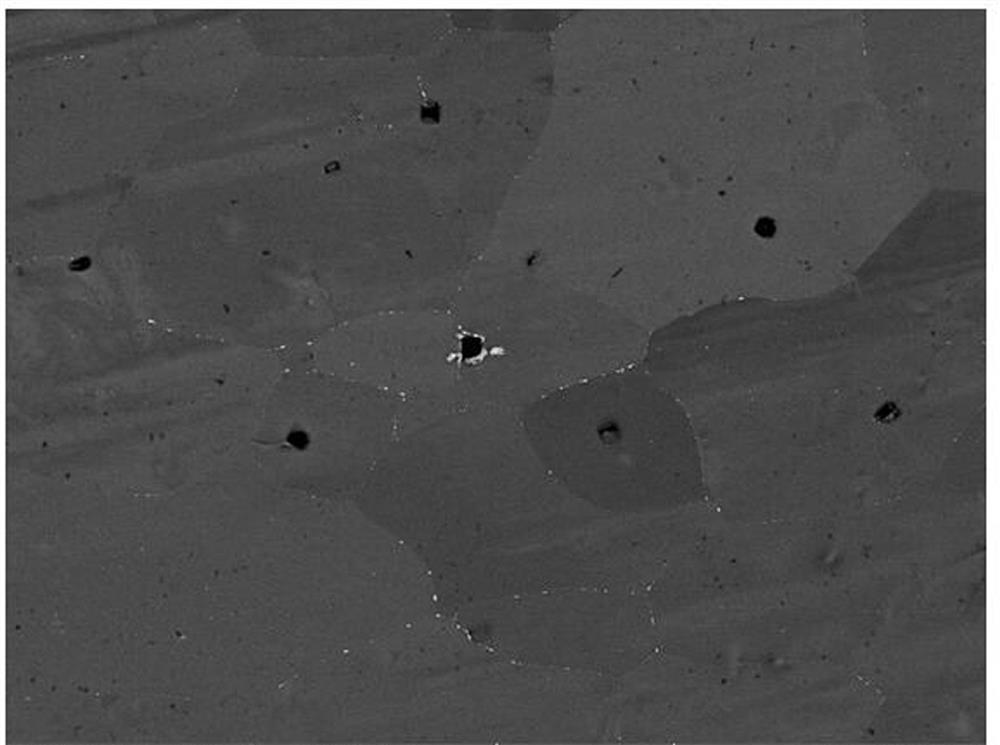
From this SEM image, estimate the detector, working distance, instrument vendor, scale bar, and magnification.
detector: BSE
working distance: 15.12 mm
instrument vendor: Tescan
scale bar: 50000 nm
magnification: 1 K X